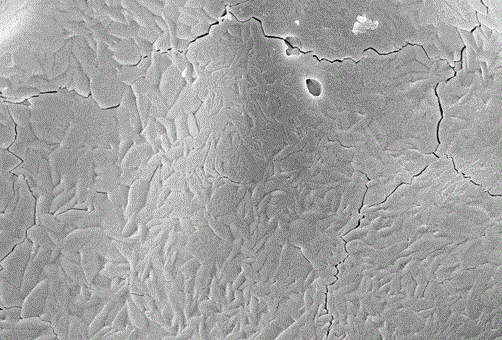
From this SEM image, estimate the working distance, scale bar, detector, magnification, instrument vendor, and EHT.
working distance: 10.6 mm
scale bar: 1000 nm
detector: InLens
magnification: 10 K X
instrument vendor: Zeiss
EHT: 5 kV